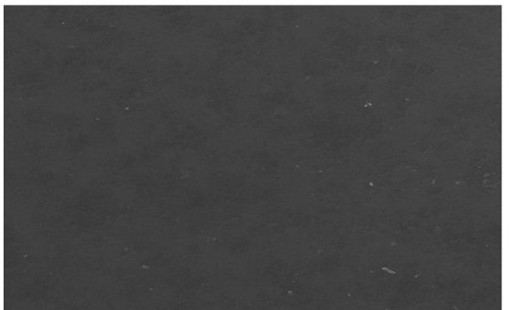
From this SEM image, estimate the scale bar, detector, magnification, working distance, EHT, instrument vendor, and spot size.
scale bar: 500000 nm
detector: ETD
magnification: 0.2 K X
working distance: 13 mm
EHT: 15 kV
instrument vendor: FEI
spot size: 5